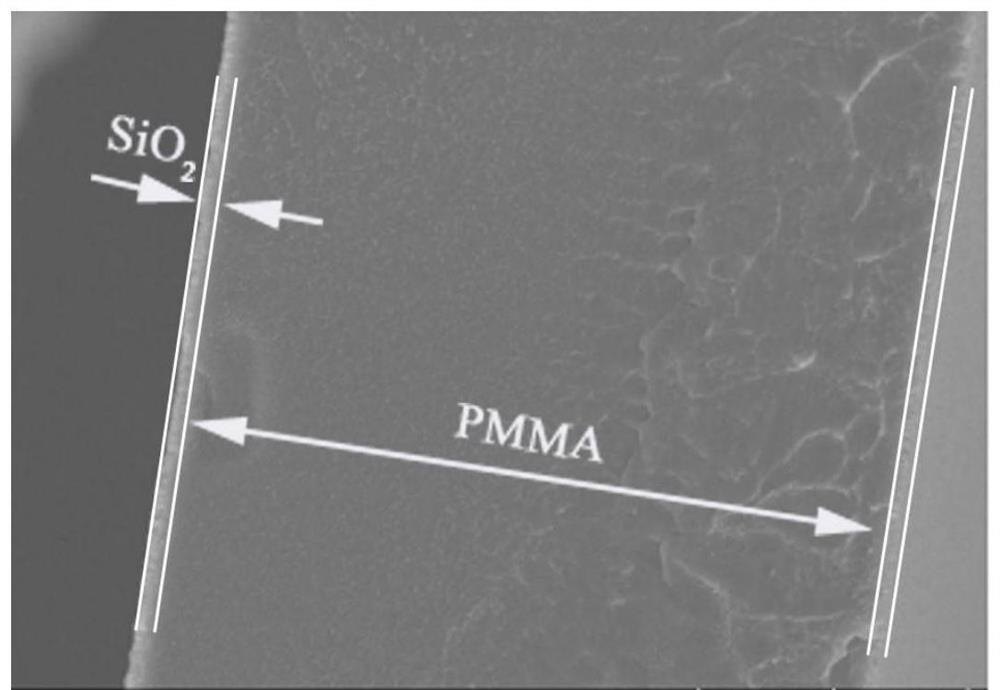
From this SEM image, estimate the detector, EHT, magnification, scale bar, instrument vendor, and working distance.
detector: SE(UL)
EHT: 5 kV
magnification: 7 K X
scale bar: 5000 nm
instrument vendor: Hitachi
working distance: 8.4 mm